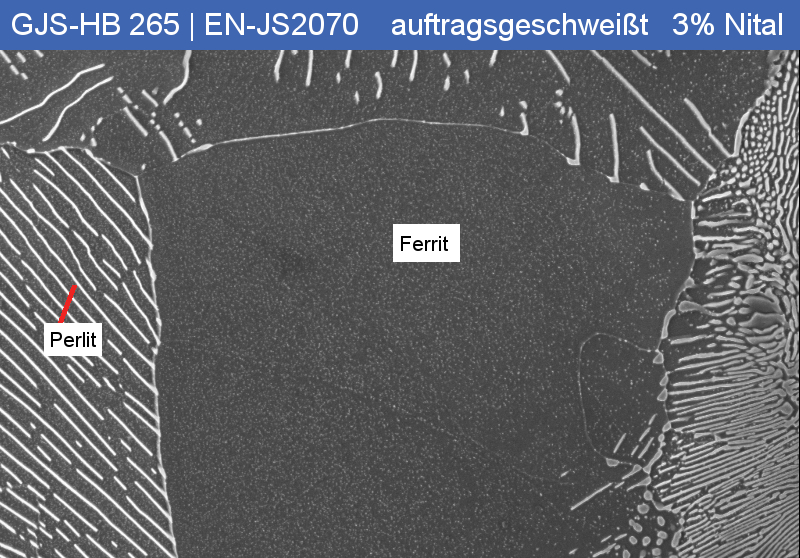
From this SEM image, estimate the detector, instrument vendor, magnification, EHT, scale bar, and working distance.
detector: InLens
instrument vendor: Zeiss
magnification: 2.5 K X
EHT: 15 kV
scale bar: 2000 nm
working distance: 5 mm